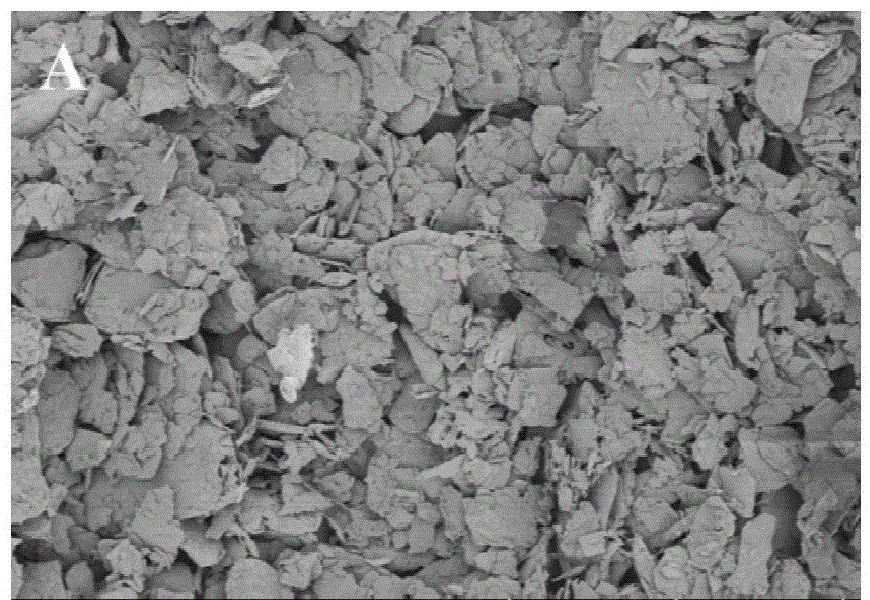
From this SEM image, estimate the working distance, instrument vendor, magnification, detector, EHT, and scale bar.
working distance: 9.2 mm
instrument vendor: Hitachi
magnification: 0.5 K X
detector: SE(UL)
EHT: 1 kV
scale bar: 100000 nm